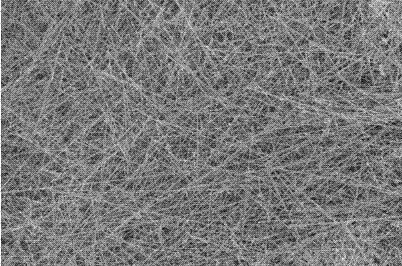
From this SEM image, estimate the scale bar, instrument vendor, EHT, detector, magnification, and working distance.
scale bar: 10000 nm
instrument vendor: Zeiss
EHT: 3 kV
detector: InLens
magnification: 1 K X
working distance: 6.3 mm